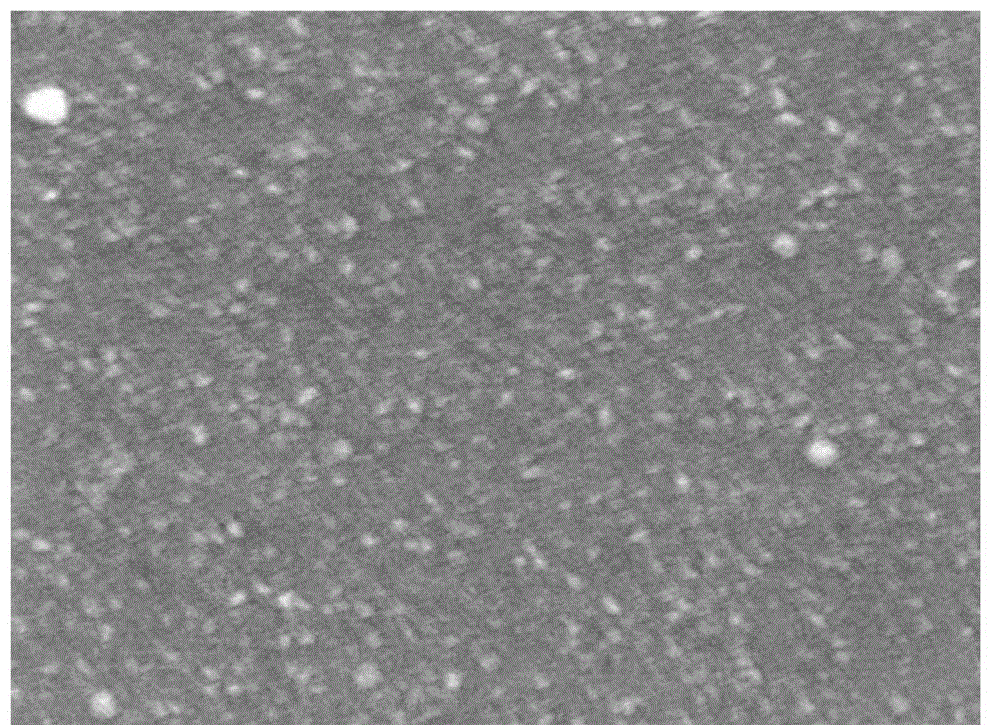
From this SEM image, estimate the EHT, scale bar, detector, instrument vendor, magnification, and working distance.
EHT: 10 kV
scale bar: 100 nm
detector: SEI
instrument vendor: JEOL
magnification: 50 K X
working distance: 13 mm